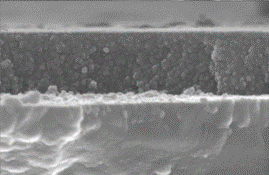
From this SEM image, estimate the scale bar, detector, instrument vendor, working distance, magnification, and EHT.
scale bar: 500 nm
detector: SE(M)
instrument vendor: Hitachi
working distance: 7.4 mm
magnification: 100 K X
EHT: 15 kV